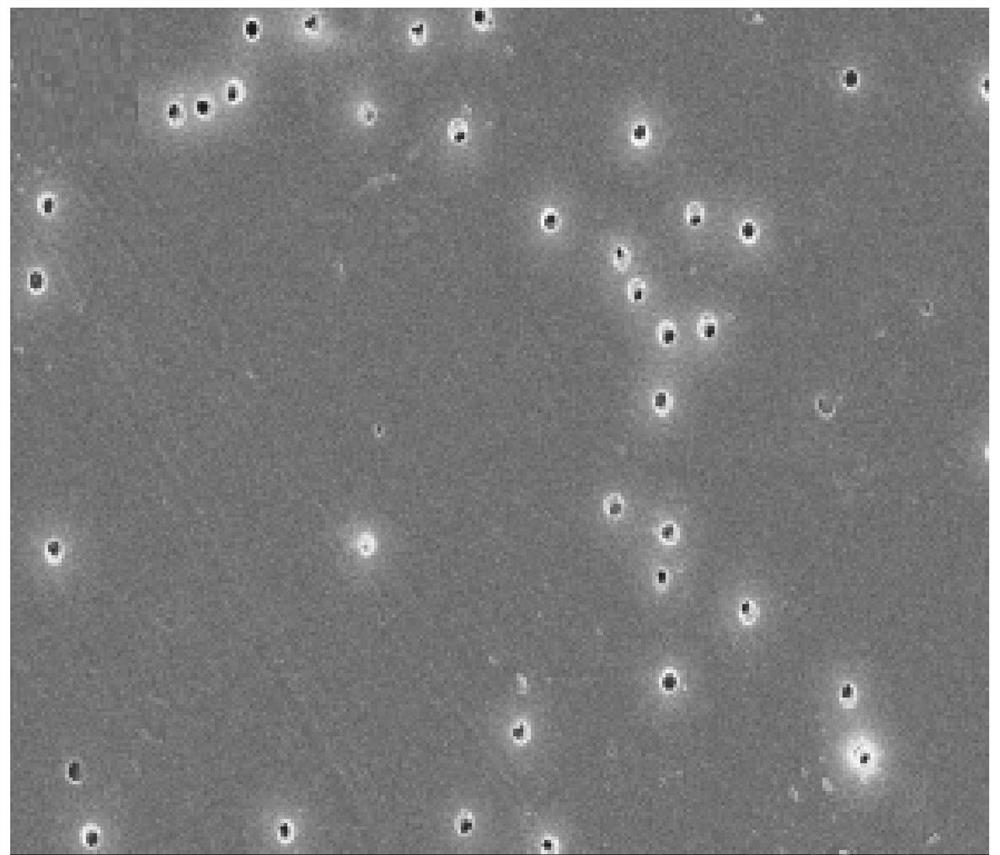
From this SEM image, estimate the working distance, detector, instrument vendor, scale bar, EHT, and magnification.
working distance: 10.4 mm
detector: SE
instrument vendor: Hitachi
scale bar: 20000 nm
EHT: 15 kV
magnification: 2 K X